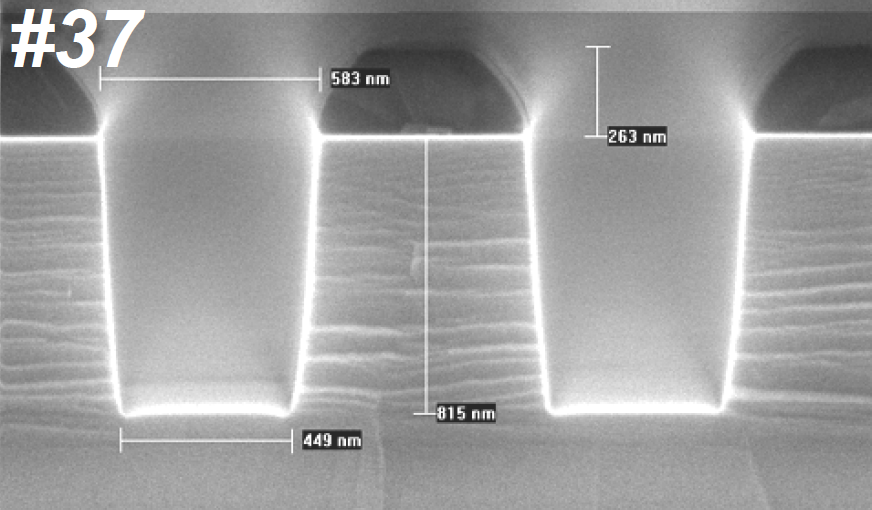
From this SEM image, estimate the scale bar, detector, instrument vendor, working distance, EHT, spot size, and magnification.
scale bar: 500 nm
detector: TLD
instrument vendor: FEI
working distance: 5 mm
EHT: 10 kV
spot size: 3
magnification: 53.504 K X